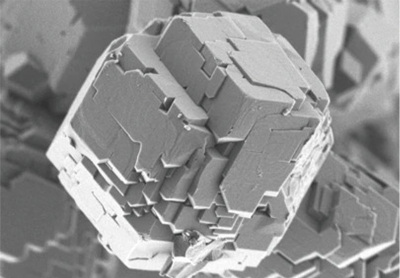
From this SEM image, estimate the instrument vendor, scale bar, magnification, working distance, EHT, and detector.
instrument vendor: Hitachi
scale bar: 2000 nm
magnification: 25 K X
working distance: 2.1 mm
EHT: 0.8 kV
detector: UD